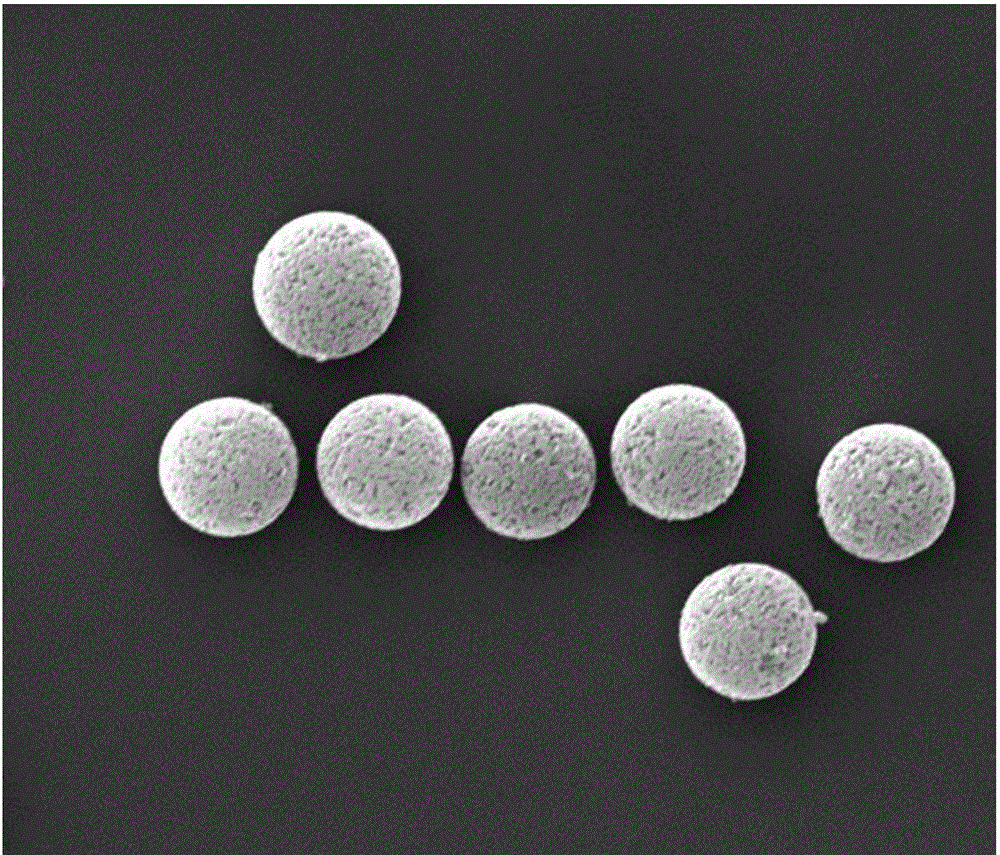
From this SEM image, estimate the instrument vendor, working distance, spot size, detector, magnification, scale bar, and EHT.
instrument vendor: FEI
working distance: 23.9 mm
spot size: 4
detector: ETD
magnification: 2 K X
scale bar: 20000 nm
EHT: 30 kV